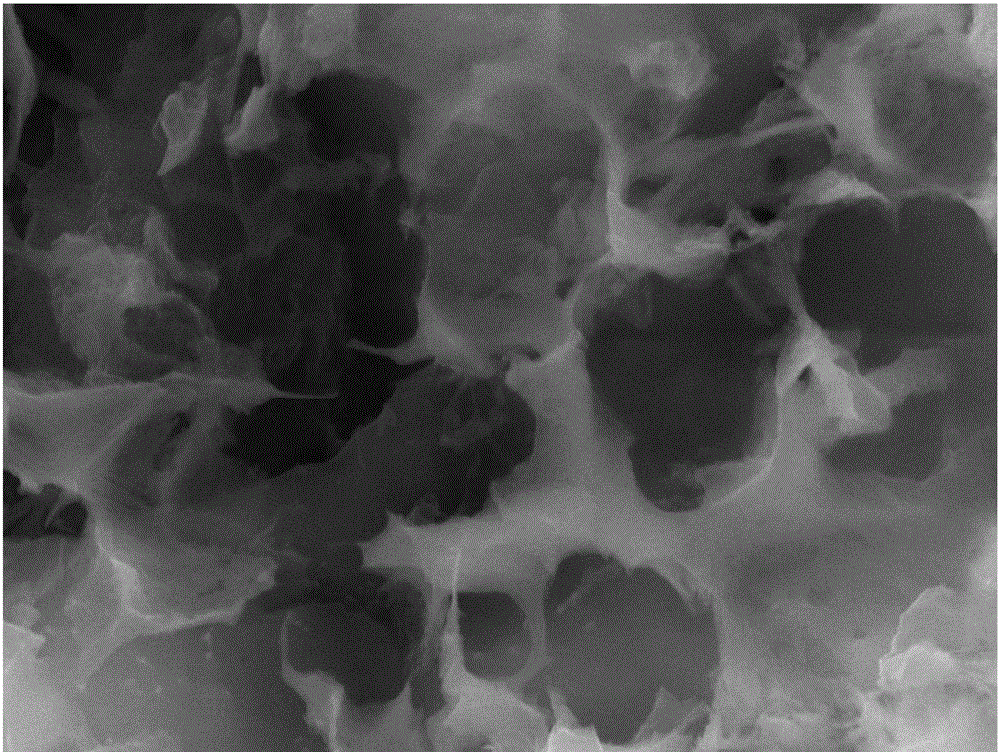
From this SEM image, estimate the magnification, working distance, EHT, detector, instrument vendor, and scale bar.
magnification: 50 K X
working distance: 14.81 mm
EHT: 20 kV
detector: SE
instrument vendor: Tescan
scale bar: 1000 nm